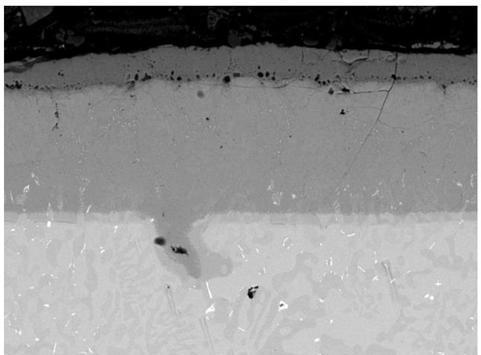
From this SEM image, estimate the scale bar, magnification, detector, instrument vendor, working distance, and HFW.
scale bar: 50000 nm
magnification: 1 K X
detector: BSE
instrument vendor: Tescan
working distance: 16.6 mm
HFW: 277 µm